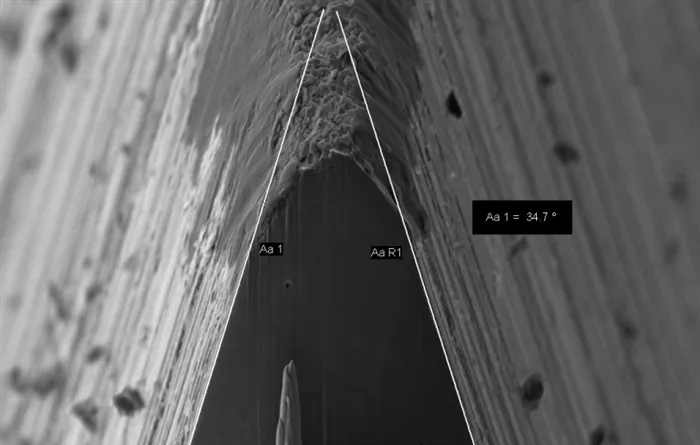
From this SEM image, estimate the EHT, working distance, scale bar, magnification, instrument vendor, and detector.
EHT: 5 kV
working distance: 6.5 mm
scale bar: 2000 nm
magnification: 3 K X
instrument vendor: Zeiss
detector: SE2